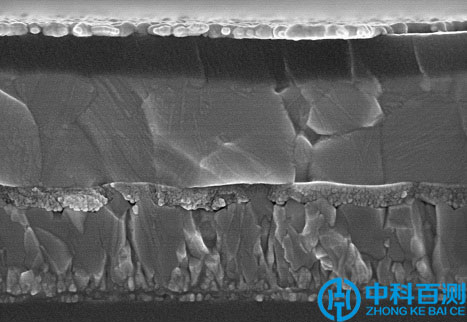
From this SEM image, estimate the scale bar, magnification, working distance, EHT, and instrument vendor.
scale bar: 1000 nm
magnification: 45 K X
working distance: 3.3 mm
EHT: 5 kV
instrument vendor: Hitachi